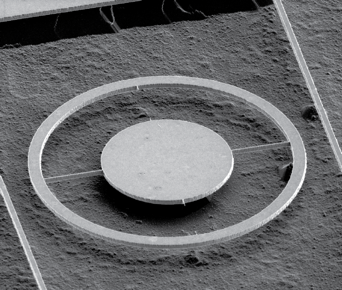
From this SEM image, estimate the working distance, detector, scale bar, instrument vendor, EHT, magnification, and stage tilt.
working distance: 4.5 mm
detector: ETD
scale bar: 20000 nm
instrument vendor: FEI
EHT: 3 kV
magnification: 2.498 K X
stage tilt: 52°